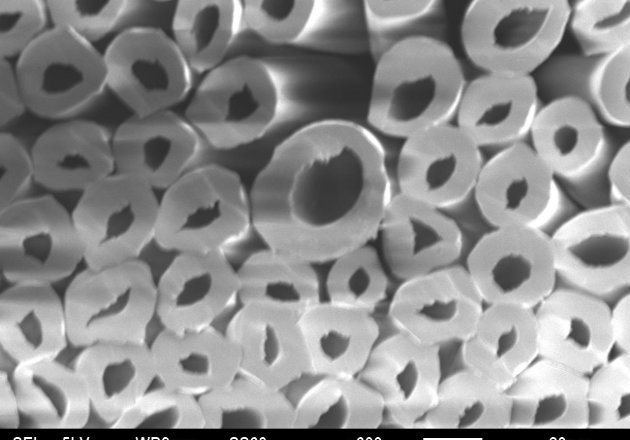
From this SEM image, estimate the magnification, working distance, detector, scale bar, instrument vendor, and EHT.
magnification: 0.6 K X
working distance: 9 mm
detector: SEI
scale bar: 20000 nm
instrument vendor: JEOL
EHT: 5 kV